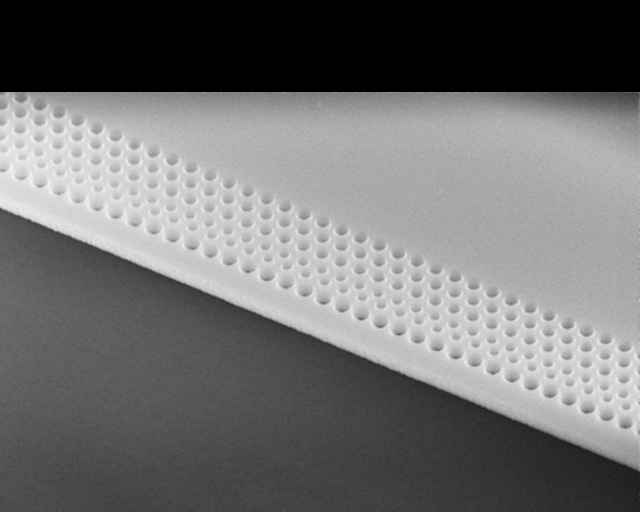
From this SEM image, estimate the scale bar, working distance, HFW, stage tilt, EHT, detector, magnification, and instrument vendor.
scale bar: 2000 nm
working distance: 4 mm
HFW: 6.23 µm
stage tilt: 45°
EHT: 10 kV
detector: TLD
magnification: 20.391 K X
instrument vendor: FEI